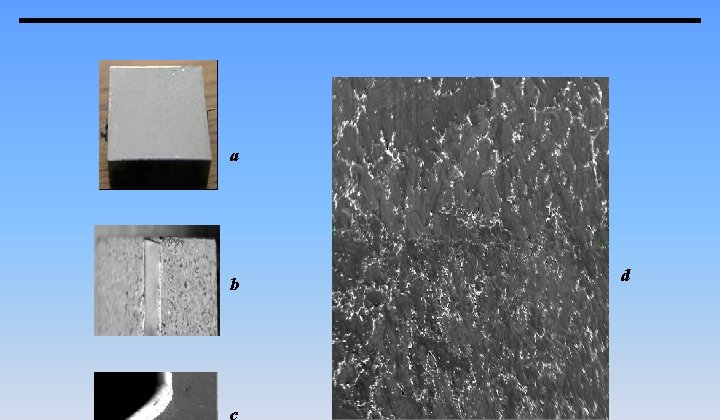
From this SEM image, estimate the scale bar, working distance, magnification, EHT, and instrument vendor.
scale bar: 1000000 nm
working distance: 25.6 mm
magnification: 0.05 K X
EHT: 20 kV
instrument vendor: JEOL